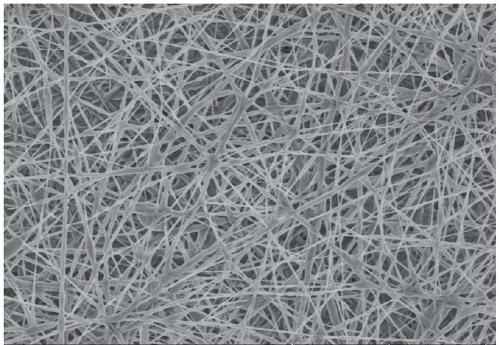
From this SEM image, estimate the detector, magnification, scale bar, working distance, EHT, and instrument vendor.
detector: SE(UL)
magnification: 5 K X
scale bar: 10000 nm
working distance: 9.3 mm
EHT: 3 kV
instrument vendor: Hitachi